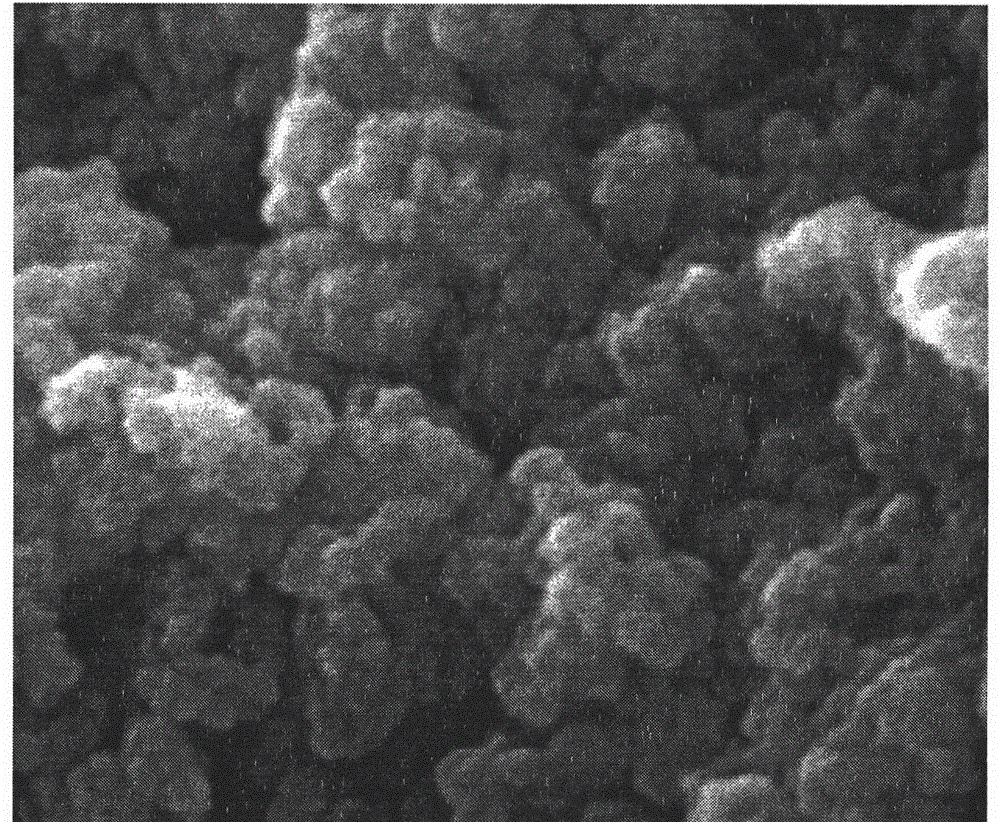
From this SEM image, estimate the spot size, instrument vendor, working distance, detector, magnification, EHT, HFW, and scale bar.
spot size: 4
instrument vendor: FEI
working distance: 8.1 mm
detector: ETD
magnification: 10 K X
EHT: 20 kV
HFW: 13.52 µm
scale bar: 2000 nm